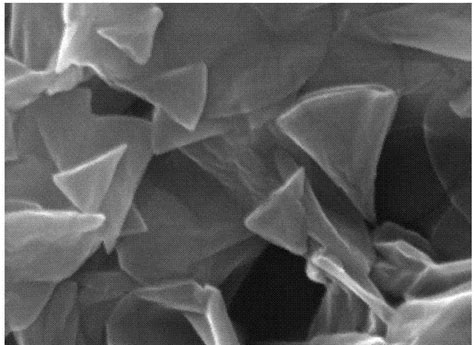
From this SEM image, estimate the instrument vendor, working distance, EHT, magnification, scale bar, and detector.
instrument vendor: Tescan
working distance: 15.76 mm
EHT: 20 kV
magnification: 100 K X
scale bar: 500 nm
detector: SE